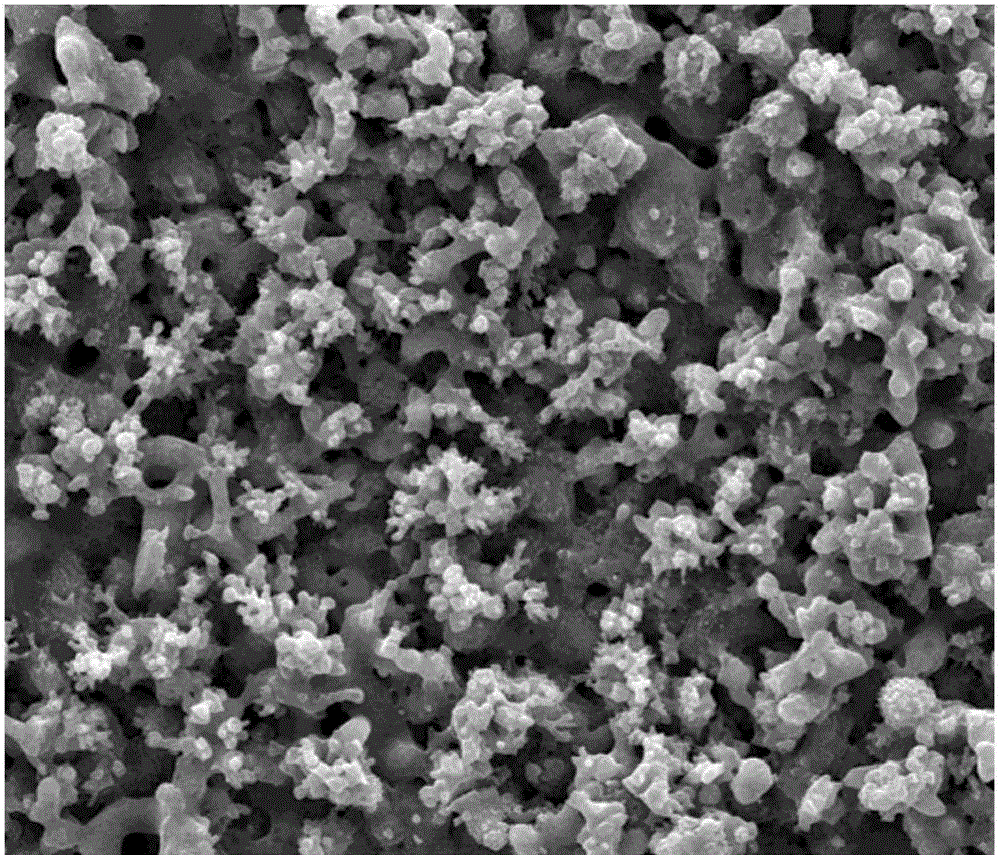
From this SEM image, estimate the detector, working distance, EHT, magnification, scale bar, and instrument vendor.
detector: SE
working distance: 10.5 mm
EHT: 30 kV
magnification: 2 K X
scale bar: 50000 nm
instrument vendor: FEI